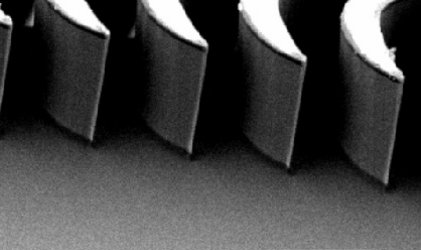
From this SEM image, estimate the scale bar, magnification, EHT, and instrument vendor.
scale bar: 50000 nm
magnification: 0.37 K X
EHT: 15 kV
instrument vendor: JEOL